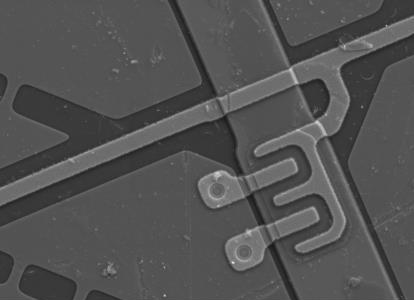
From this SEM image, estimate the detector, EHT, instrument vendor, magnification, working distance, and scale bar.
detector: SE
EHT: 15 kV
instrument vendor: FEI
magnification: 0.691 K X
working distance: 10.8983 mm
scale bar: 40000 nm